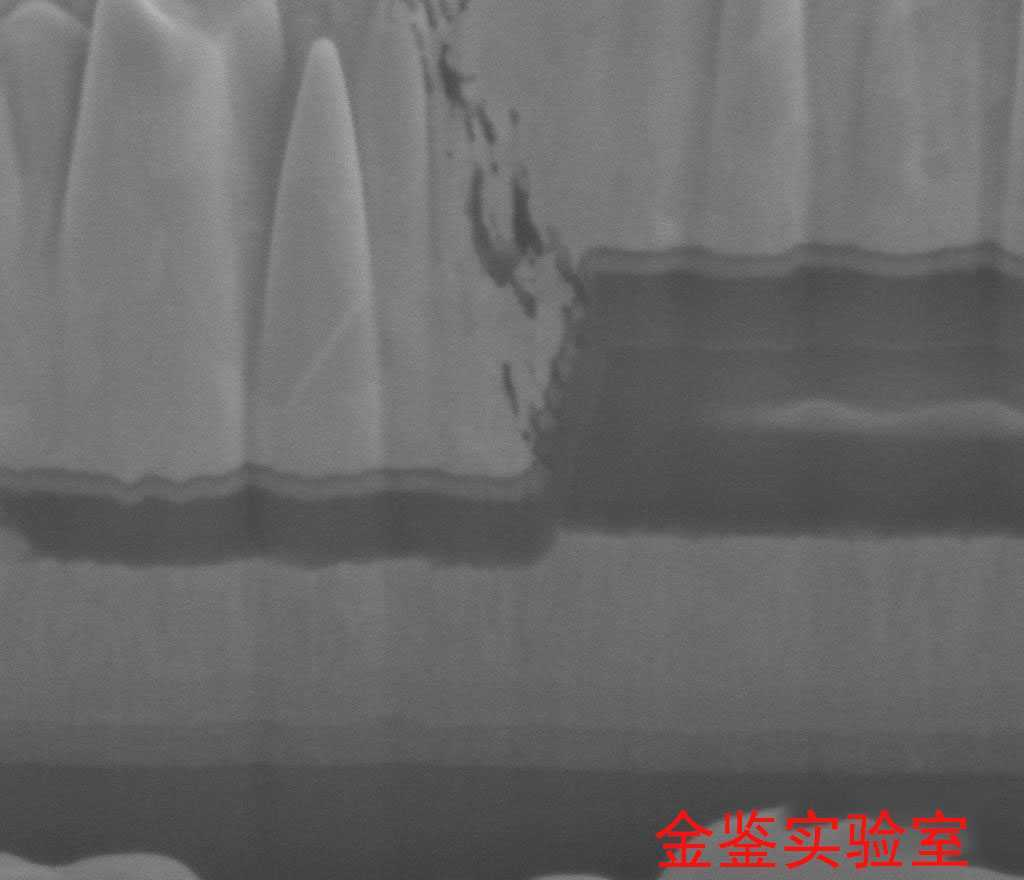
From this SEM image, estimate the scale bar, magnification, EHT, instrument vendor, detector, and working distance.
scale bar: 1000 nm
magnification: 80 K X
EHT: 5 kV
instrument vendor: FEI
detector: ETD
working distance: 4.9 mm